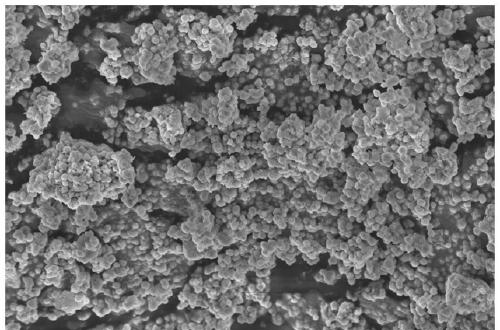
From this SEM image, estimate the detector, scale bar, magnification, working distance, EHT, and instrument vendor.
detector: InLens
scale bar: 1000 nm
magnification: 5 K X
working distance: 6.9 mm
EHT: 5 kV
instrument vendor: Zeiss